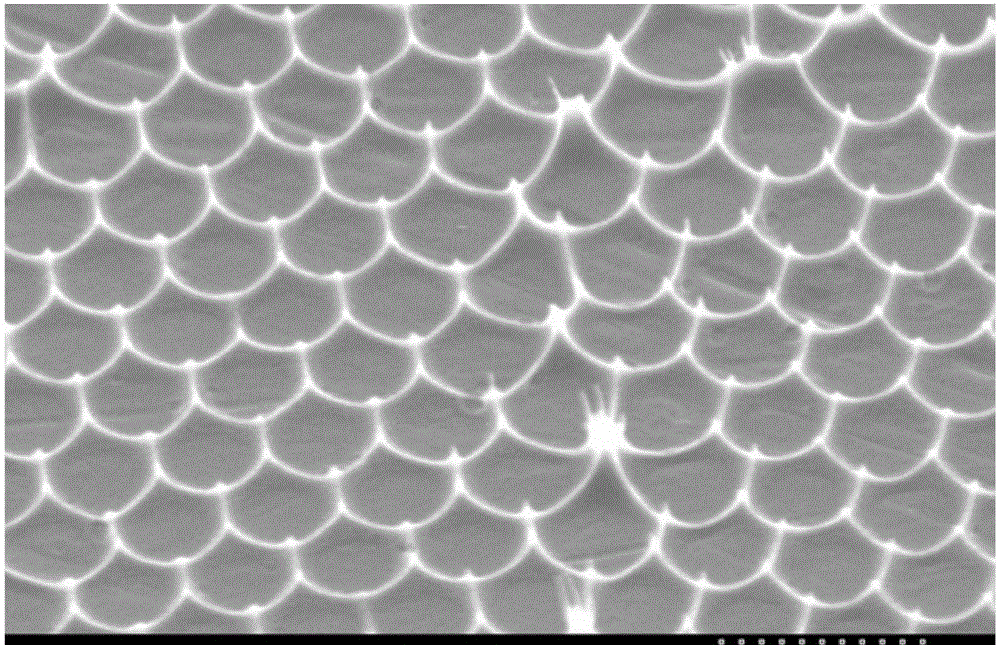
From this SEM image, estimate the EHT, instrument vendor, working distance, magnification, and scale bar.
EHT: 15 kV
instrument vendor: Hitachi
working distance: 20.9 mm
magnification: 2.7 K X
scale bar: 10000 nm